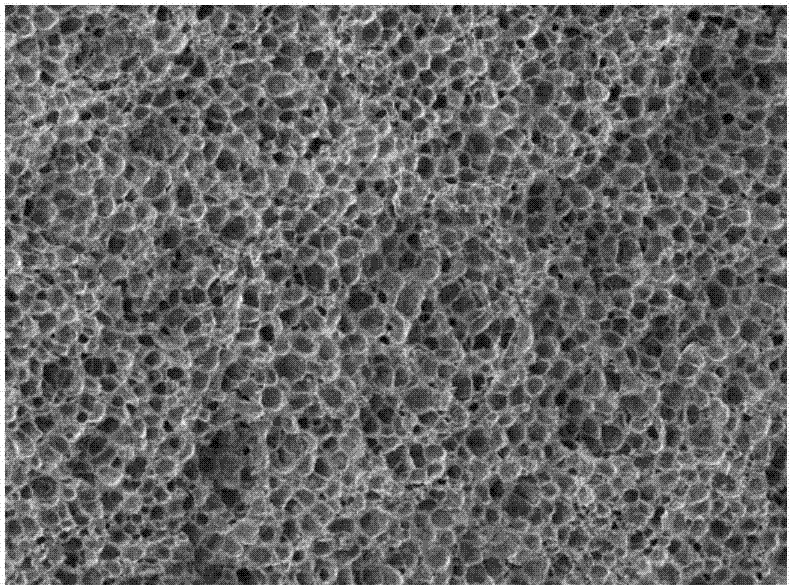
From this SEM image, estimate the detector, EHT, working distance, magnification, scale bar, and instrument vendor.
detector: SEI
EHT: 5 kV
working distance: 13 mm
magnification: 0.1 K X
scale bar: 100000 nm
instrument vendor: JEOL